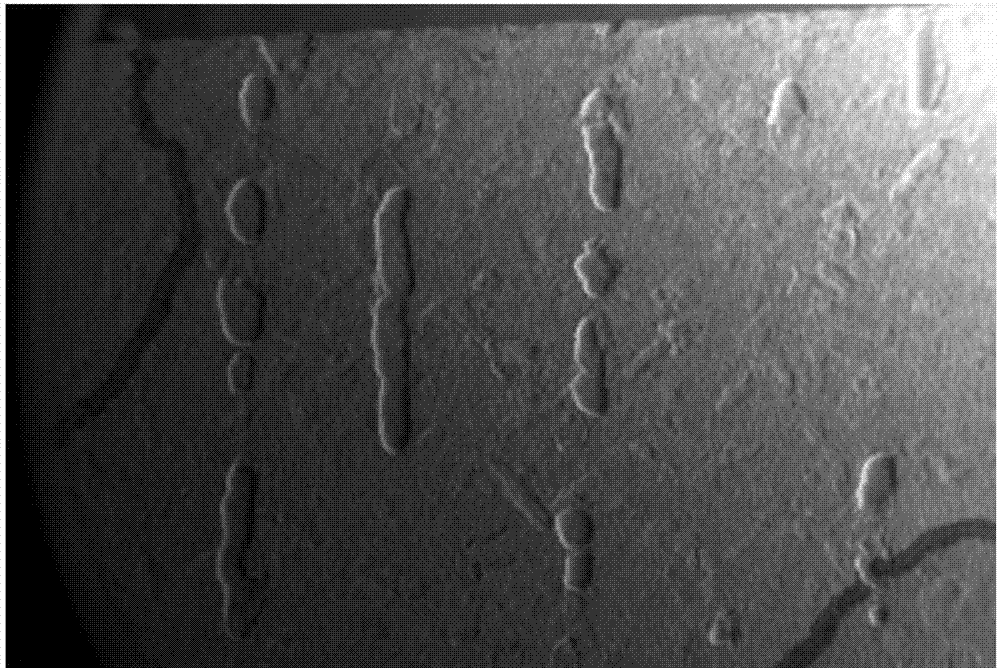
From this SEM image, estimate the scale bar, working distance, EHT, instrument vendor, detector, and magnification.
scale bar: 200000 nm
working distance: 9.5 mm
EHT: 20 kV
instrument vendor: Zeiss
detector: SE1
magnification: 0.021 K X